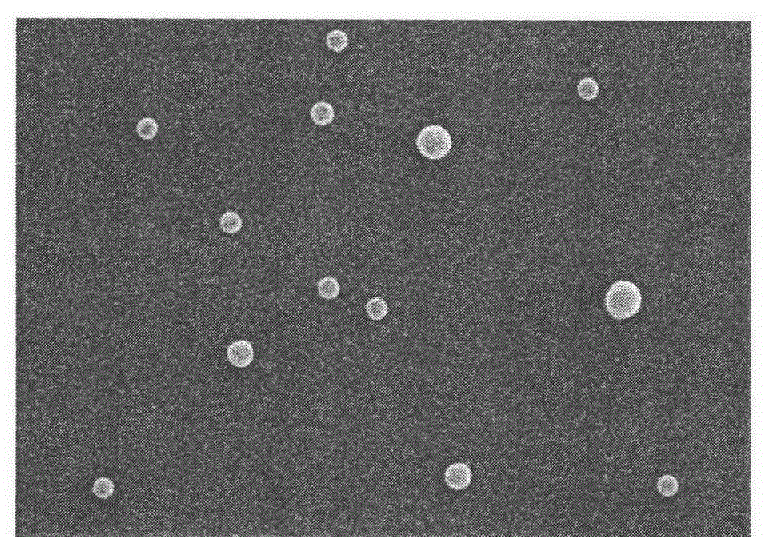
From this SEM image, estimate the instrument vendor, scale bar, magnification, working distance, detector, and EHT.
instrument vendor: Hitachi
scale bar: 3000 nm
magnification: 16 K X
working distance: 7 mm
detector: SE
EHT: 15 kV